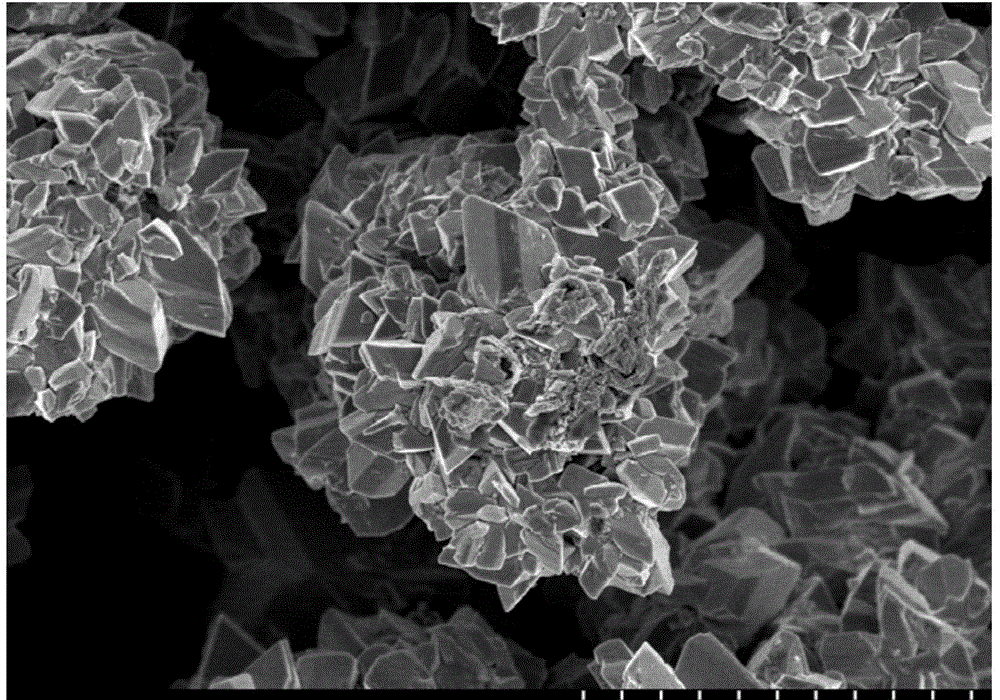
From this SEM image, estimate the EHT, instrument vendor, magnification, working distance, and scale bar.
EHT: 3 kV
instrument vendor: Hitachi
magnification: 10 K X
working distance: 8.7 mm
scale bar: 5000 nm